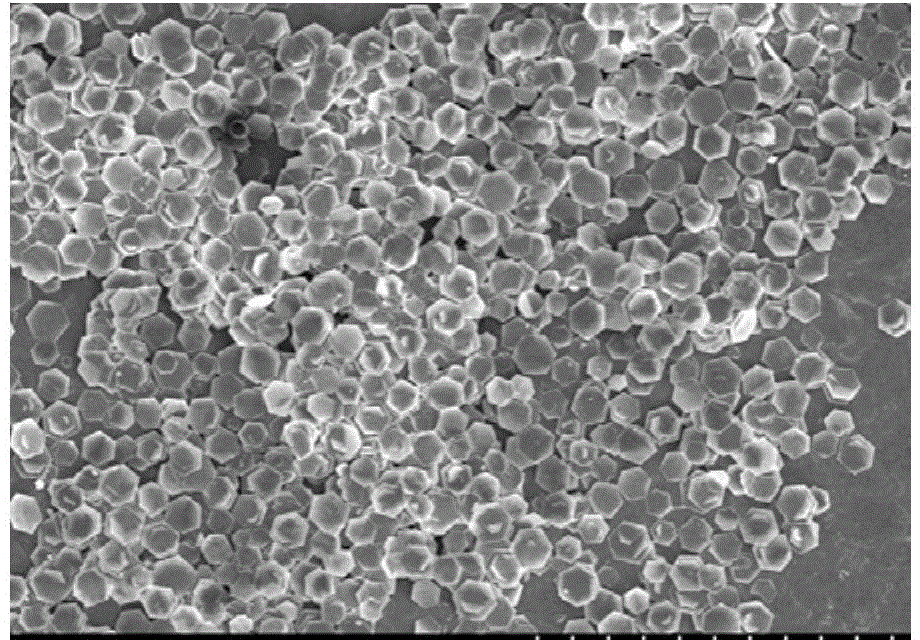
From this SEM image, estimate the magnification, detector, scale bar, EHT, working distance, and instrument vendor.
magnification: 1 K X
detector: SE(M)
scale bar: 50000 nm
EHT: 5 kV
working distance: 7.3 mm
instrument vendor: Hitachi